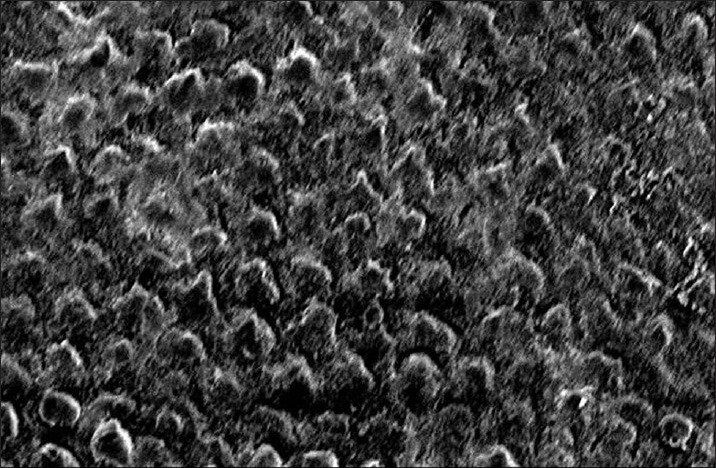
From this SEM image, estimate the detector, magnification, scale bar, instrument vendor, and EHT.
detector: SEI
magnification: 1.6 K X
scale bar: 10000 nm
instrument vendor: JEOL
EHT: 5 kV